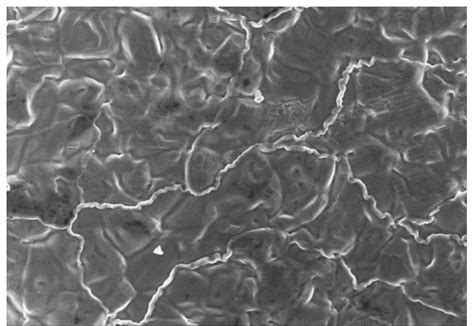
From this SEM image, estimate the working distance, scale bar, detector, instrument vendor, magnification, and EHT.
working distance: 7.9 mm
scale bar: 50000 nm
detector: SE(UL)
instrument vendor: Hitachi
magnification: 1 K X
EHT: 15 kV